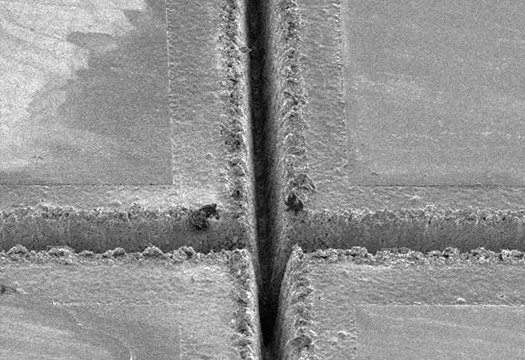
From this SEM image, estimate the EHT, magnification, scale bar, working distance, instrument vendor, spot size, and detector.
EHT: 5 kV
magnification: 0.2 K X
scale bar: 100000 nm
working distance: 10.6 mm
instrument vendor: FEI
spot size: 3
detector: SE